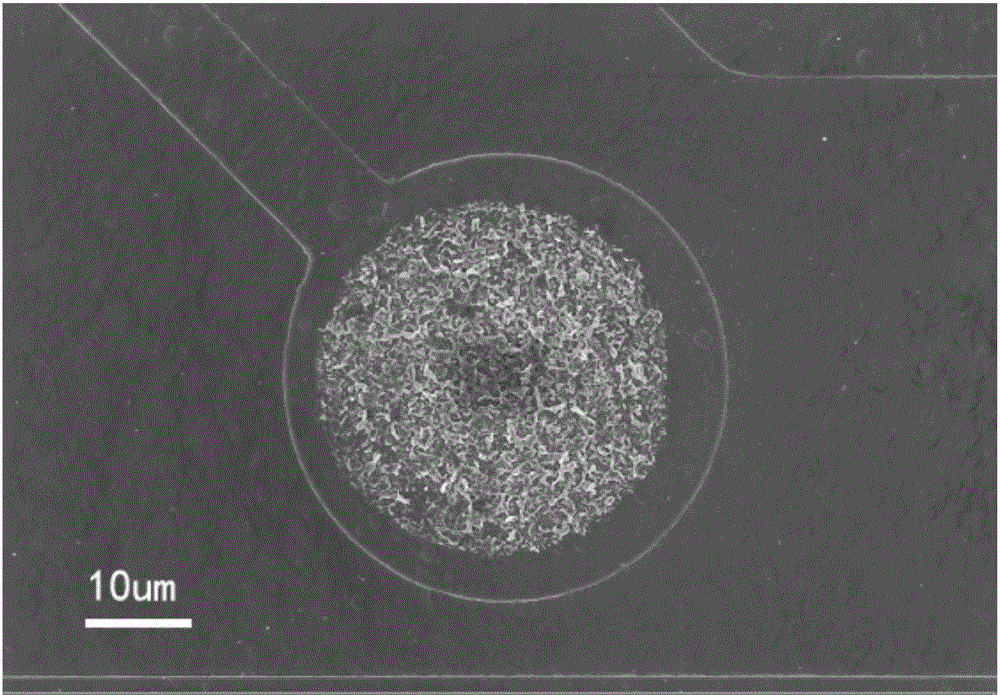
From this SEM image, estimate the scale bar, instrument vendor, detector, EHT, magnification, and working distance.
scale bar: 2000 nm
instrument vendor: Zeiss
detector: InLens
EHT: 5 kV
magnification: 1 K X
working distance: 9 mm